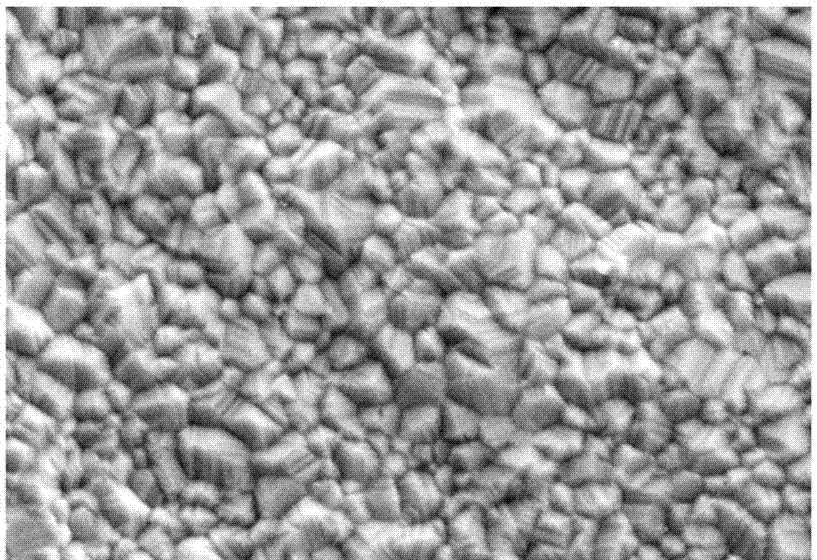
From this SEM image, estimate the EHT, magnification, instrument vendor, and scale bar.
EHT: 20 kV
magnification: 3 K X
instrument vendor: other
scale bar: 10000 nm